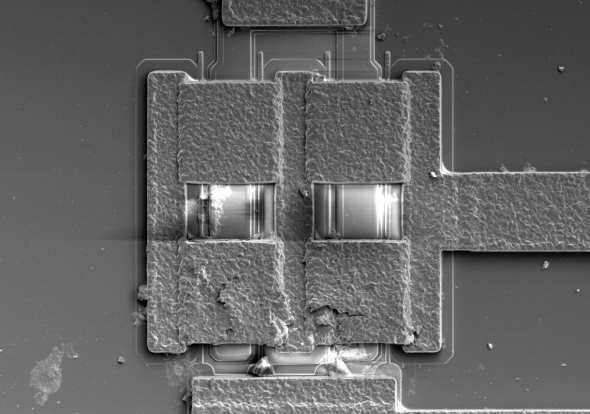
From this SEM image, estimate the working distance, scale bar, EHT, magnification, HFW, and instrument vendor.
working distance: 4.2 mm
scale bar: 50000 nm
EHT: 2 kV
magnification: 2.5 K X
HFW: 166 µm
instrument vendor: FEI